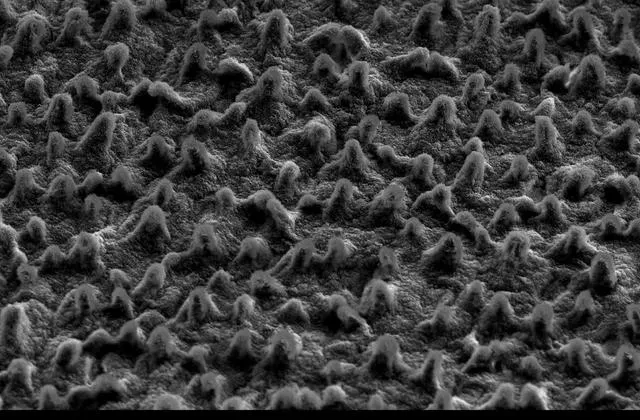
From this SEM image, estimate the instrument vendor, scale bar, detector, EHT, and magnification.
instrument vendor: JEOL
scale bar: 50000 nm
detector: SEI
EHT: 15 kV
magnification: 0.5 K X